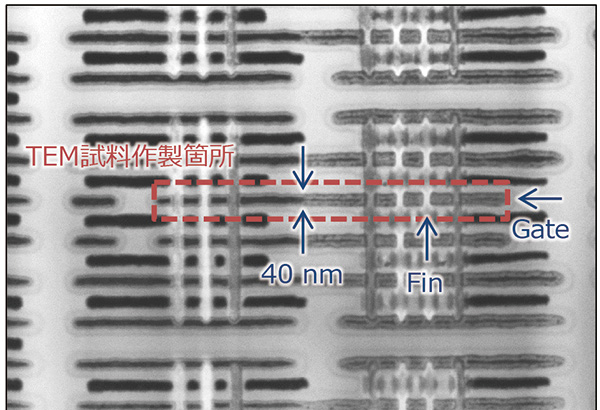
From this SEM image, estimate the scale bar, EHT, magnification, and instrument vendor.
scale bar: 100 nm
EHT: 30 kV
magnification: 100 K X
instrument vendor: JEOL